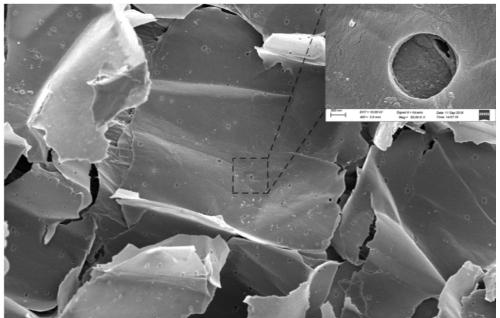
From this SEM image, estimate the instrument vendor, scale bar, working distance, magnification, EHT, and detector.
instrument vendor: Zeiss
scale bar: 20000 nm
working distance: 3.9 mm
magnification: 1 K X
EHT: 10 kV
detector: InLens